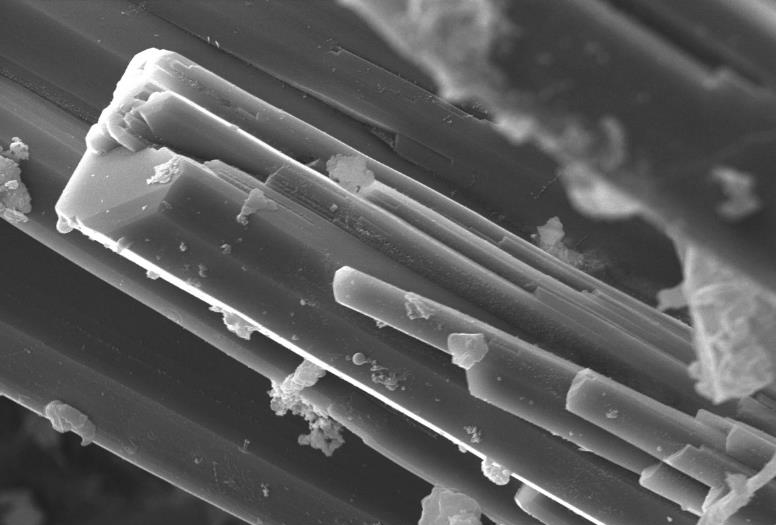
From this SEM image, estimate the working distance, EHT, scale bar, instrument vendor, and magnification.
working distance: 10.1 mm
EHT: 15 kV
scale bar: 7000 nm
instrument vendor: other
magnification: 3.488 K X